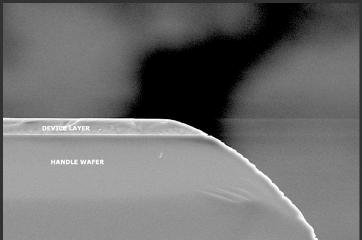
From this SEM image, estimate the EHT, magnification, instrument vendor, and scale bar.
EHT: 30 kV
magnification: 0.3 K X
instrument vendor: JEOL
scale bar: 33300 nm